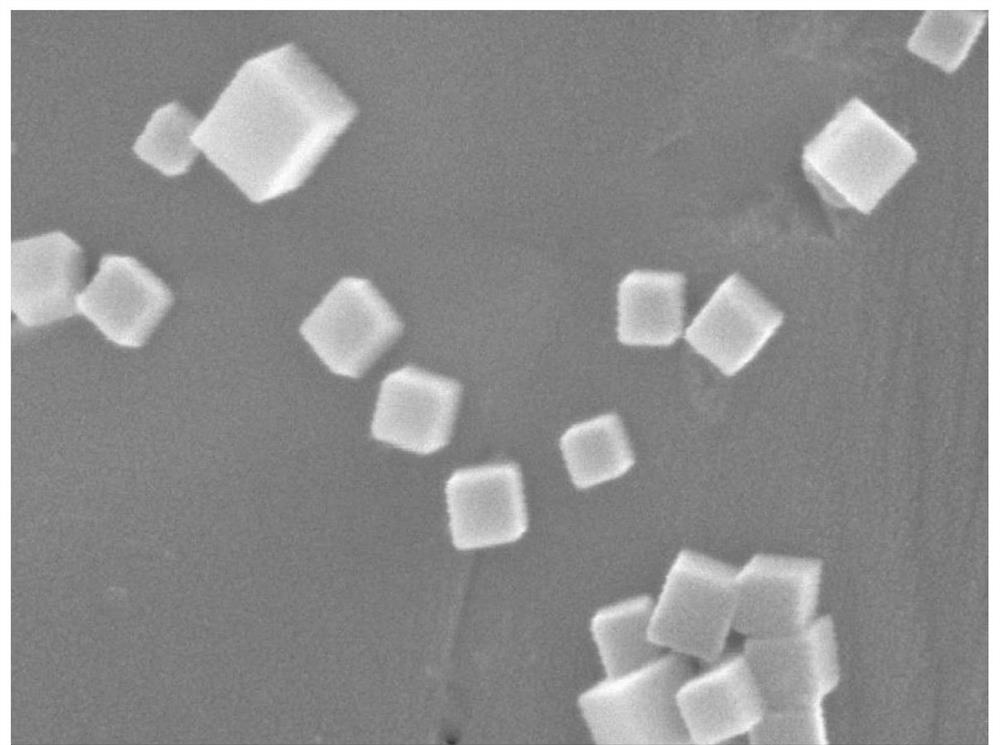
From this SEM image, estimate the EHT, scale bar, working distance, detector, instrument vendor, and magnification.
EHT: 5 kV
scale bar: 100 nm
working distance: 7.9 mm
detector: SEI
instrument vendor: JEOL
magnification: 100 K X